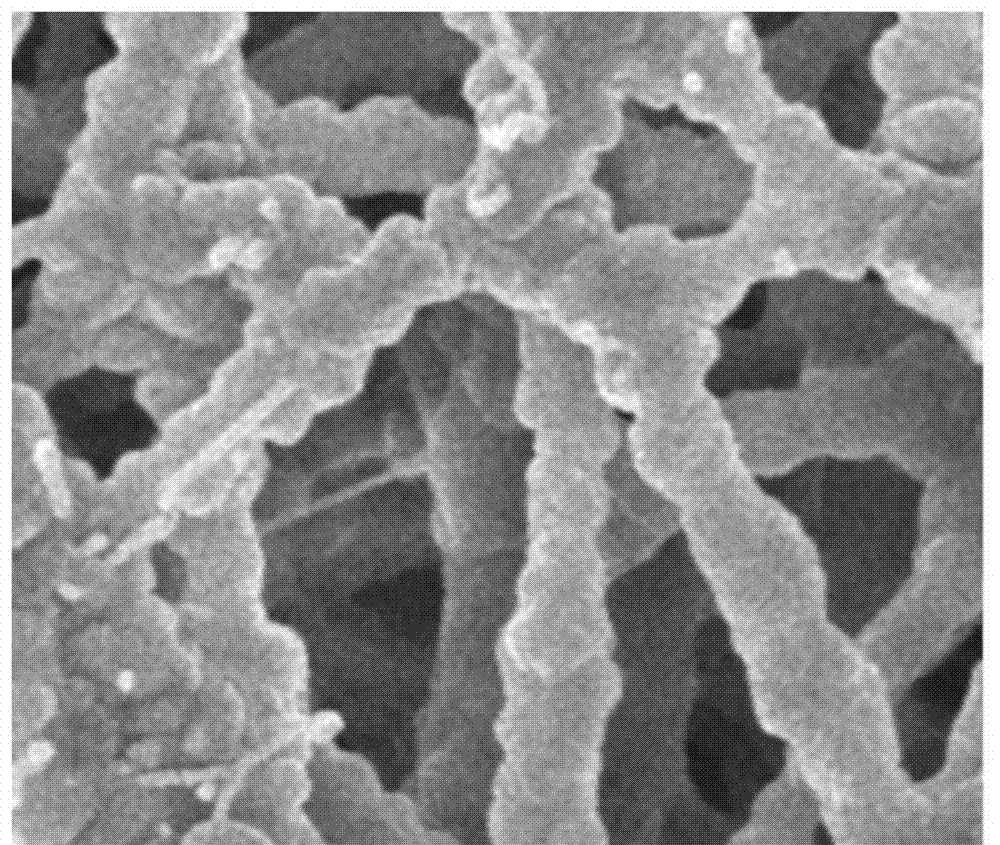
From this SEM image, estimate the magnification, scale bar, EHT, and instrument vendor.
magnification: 10 K X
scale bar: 1000 nm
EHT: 5 kV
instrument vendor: JEOL